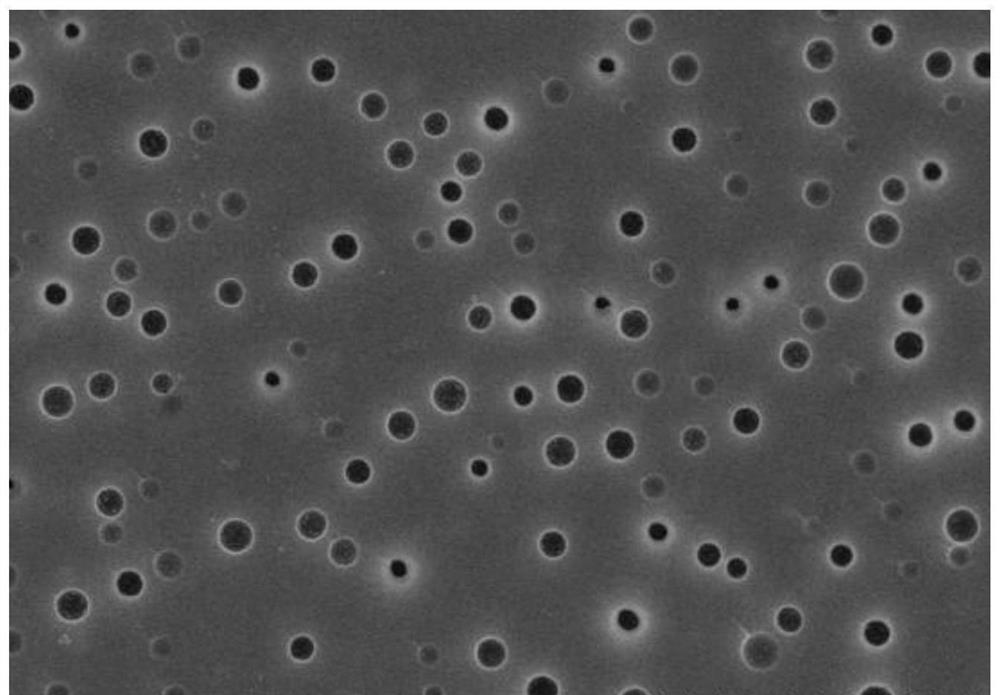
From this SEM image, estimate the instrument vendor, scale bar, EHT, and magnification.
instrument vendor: Hitachi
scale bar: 2000 nm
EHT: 5 kV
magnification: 20 K X